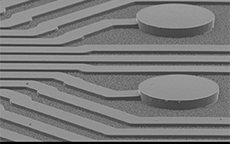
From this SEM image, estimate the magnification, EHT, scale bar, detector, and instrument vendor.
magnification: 0.1 K X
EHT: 10 kV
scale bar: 100000 nm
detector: SEI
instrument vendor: JEOL